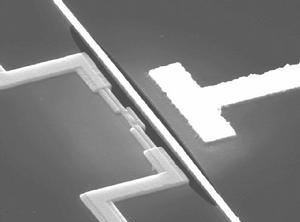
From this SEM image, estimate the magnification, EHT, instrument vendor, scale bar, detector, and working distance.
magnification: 14 K X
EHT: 15 kV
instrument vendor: JEOL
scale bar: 1000 nm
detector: SEI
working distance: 20 mm